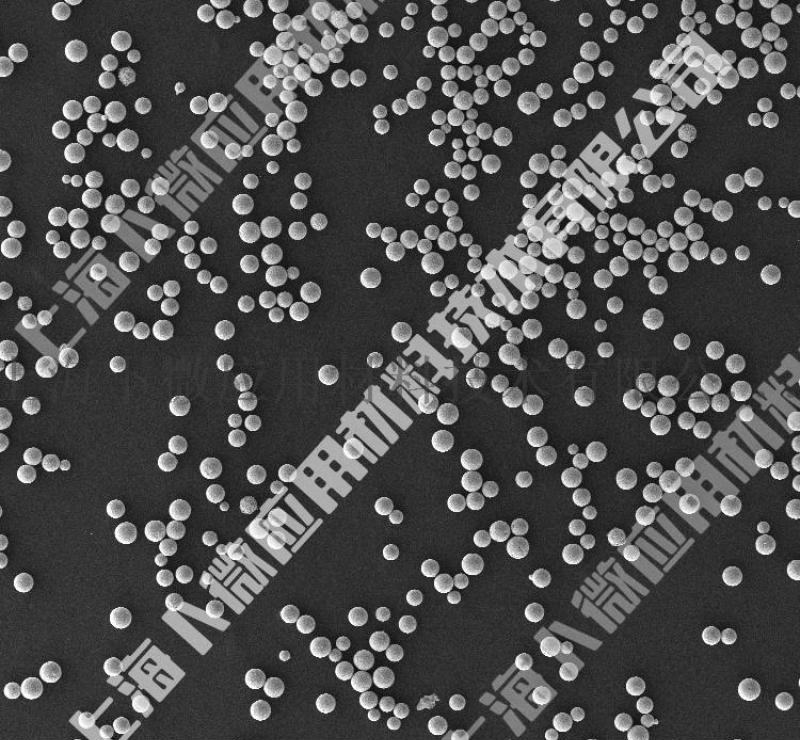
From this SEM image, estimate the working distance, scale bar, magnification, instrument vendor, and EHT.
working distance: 8.9 mm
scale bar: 100000 nm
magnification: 0.1 K X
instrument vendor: Zeiss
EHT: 10 kV